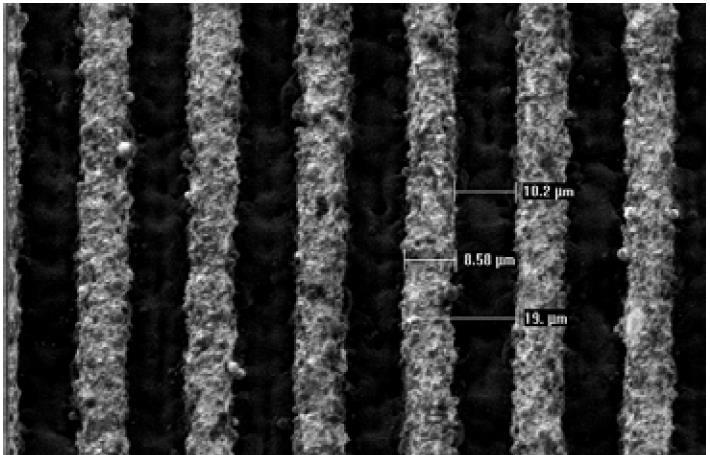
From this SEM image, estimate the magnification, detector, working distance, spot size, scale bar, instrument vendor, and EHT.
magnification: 1 K X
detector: SE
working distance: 10.3 mm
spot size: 3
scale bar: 20000 nm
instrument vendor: FEI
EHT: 1.1 kV